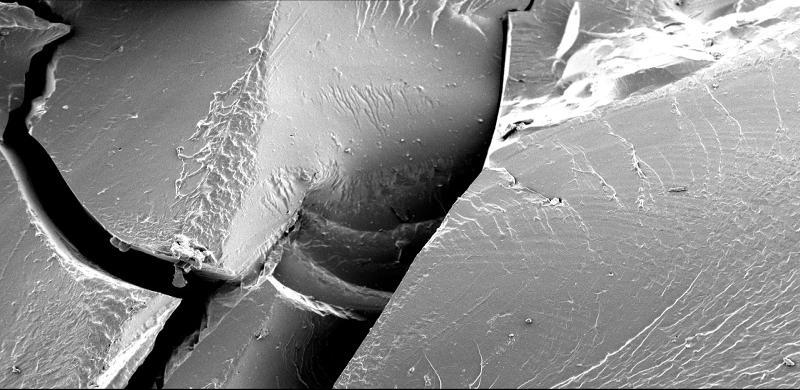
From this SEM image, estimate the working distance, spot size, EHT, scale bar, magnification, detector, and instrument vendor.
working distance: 11 mm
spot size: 50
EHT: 5 kV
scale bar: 100000 nm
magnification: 0.1 K X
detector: SEI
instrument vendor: JEOL